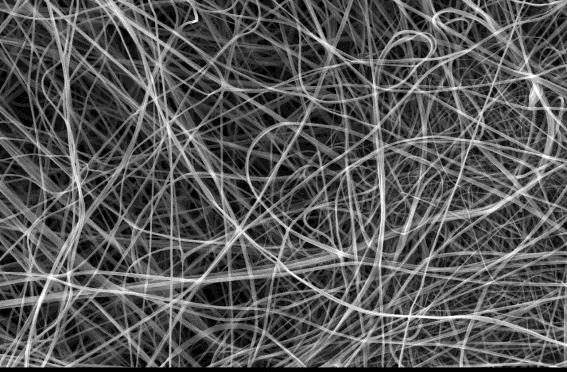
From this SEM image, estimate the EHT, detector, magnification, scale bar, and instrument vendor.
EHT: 15 kV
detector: SEI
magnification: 1 K X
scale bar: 10000 nm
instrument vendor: JEOL